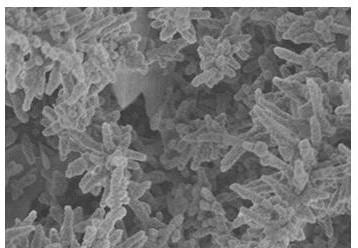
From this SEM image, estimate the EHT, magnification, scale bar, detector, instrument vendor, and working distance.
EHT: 3 kV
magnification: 10 K X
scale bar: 5000 nm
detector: SE(M)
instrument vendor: Hitachi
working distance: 9.4 mm